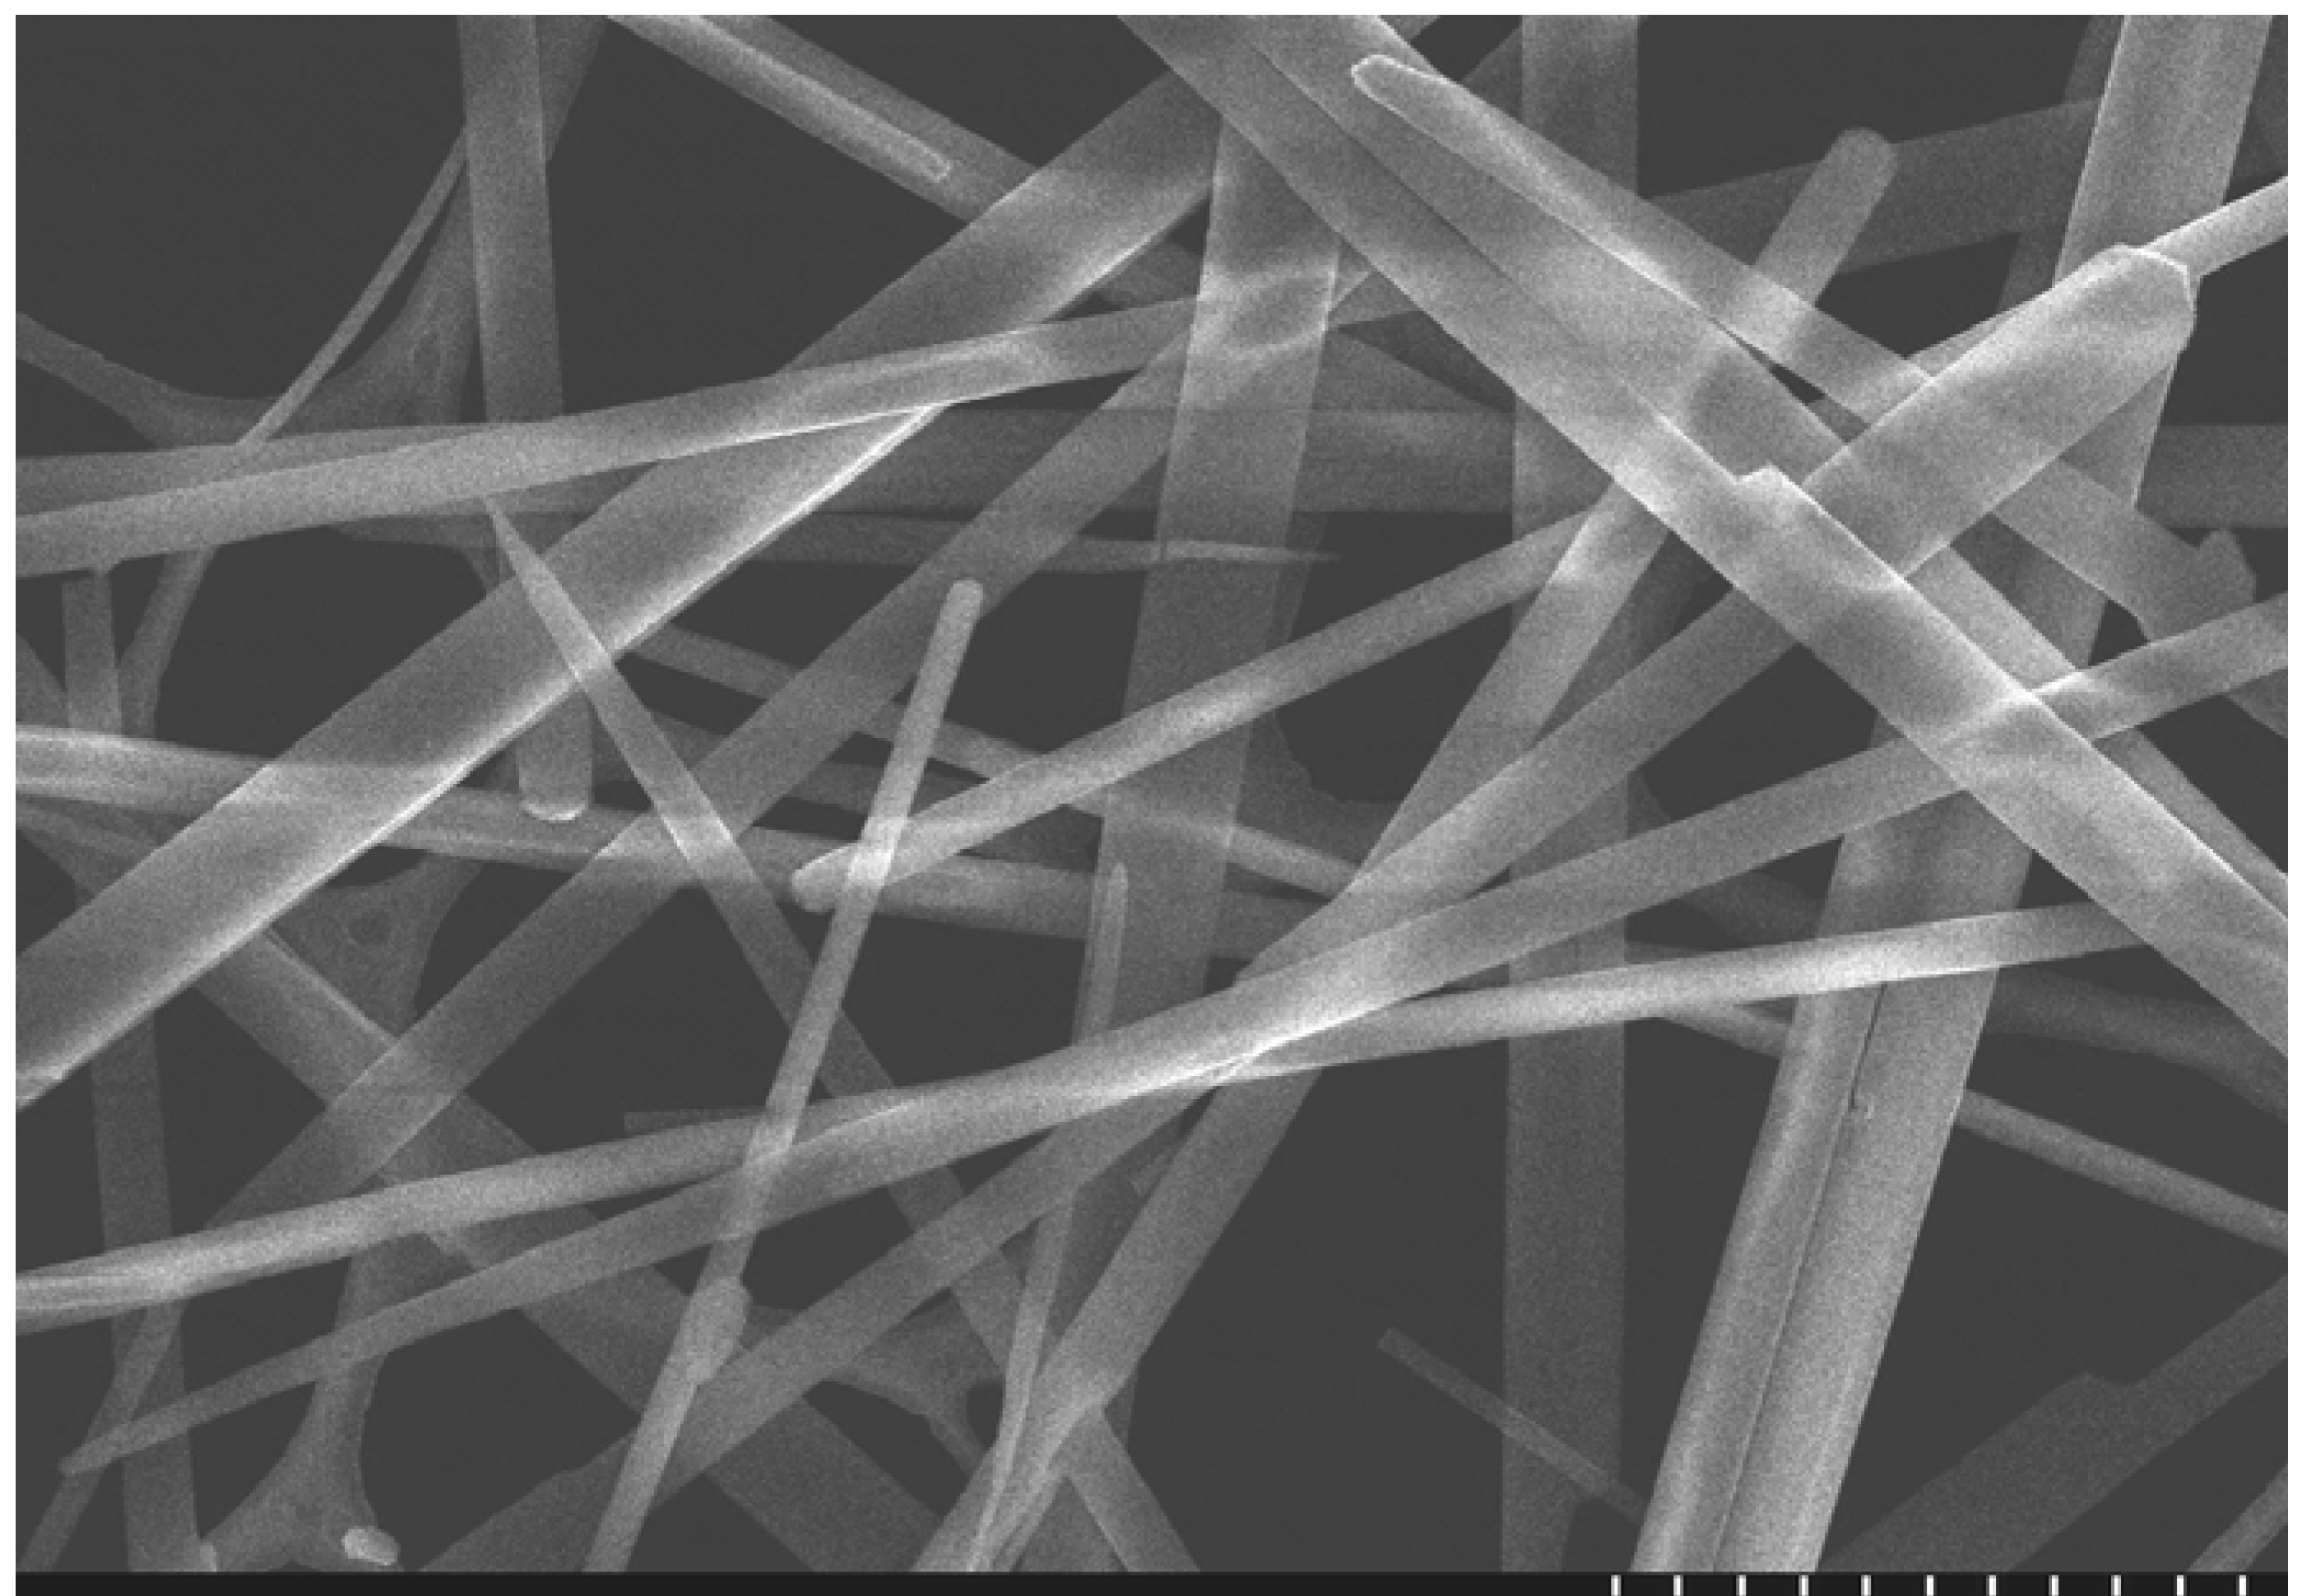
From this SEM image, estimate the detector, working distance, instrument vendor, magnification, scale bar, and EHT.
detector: SE(U)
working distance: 8.6 mm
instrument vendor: Hitachi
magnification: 7 K X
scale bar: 5000 nm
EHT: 5 kV